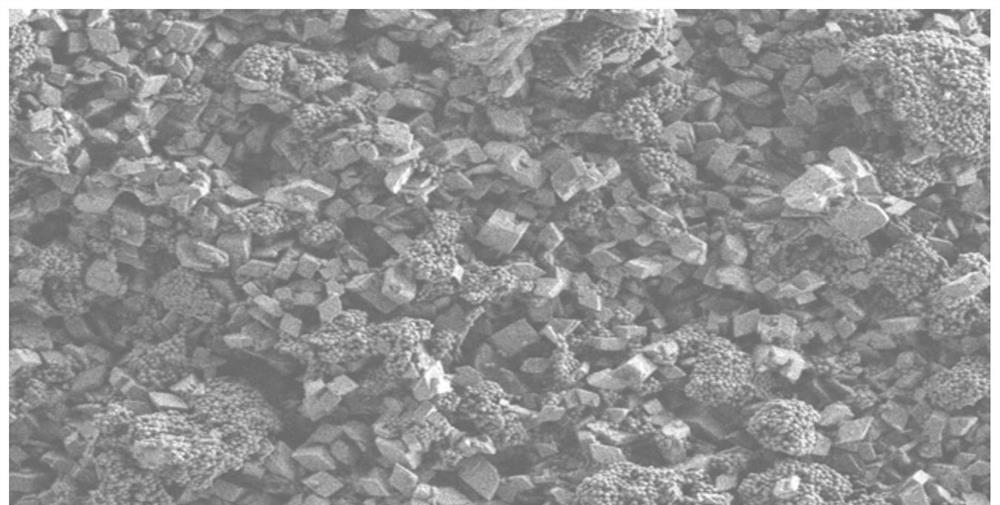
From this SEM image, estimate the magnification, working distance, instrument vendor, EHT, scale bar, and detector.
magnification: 10 K X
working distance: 3.4 mm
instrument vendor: Zeiss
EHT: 1 kV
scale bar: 1000 nm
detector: SE2